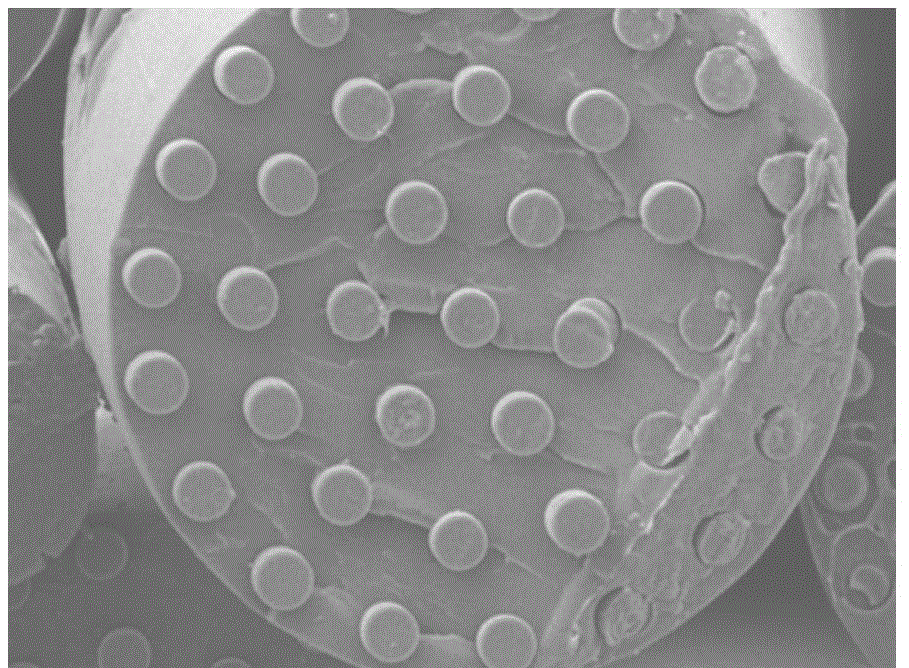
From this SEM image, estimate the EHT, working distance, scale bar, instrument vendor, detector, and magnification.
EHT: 10 kV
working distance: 8 mm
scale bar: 1000 nm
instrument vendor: JEOL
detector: SEI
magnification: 5 K X